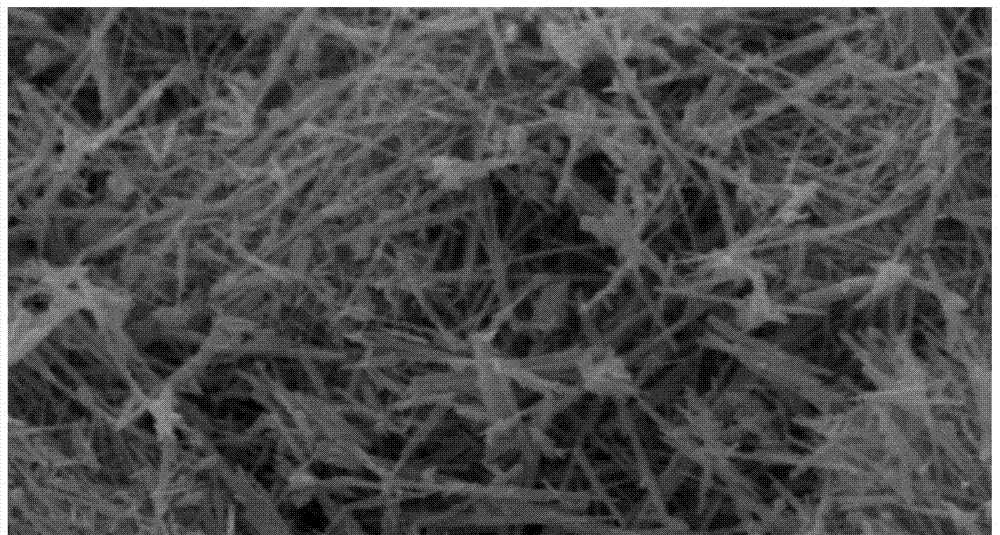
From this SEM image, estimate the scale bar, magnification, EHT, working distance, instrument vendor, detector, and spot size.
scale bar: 2000 nm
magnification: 30 K X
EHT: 15 kV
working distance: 17.5 mm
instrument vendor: FEI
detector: SE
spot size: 2.5